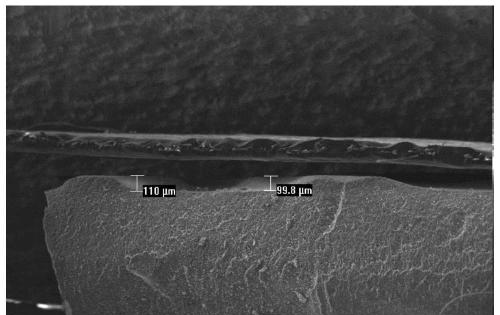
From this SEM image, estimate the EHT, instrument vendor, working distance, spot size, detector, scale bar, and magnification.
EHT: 20 kV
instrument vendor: FEI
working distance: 10.9 mm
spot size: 5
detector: SE2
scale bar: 1000000 nm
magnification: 0.037 K X